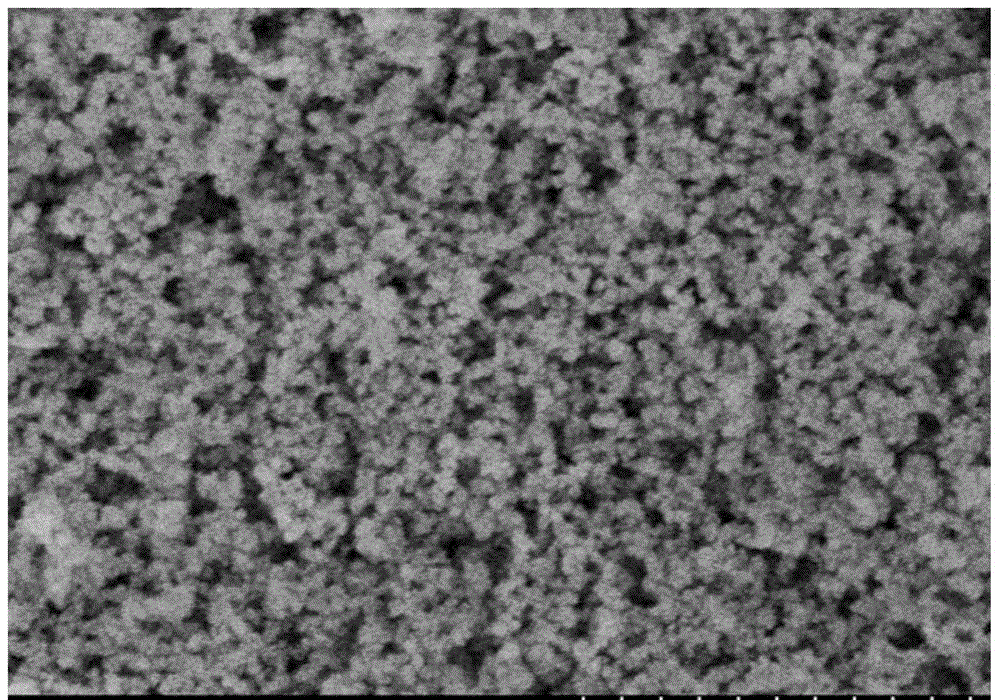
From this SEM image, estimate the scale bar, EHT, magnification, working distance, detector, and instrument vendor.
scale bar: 1000 nm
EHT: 3 kV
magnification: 50 K X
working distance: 8.6 mm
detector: SE(M)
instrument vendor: Hitachi